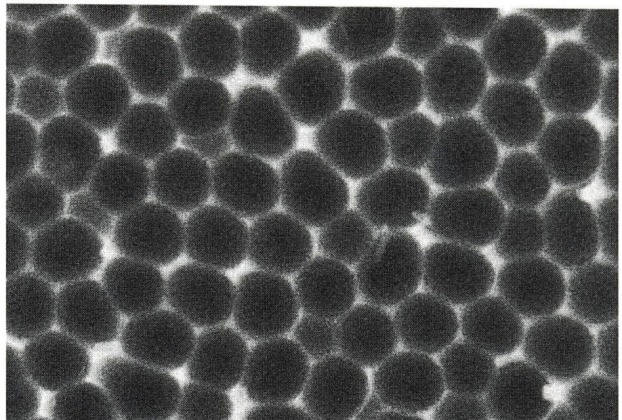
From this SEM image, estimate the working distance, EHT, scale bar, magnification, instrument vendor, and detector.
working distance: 0.5 mm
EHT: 5 kV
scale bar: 1000 nm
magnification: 50.1 K X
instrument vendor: Hitachi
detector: SE